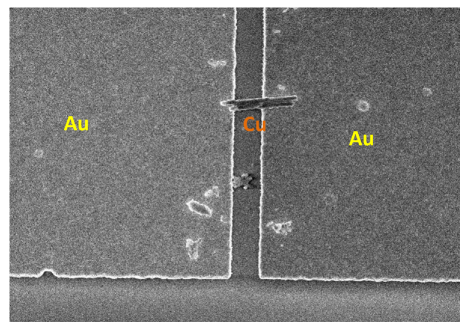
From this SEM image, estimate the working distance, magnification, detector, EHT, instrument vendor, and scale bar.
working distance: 7.8 mm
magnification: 20 K X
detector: SE(U)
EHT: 2 kV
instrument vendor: Hitachi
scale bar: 2000 nm